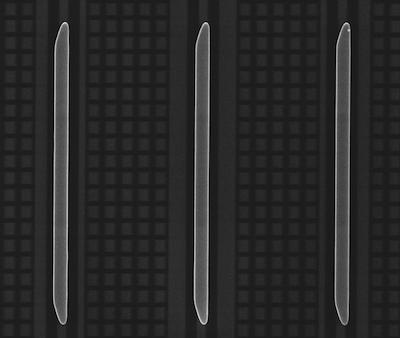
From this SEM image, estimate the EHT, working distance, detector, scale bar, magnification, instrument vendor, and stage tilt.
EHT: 18 kV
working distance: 5 mm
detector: TLD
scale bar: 20000 nm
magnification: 1.75 K X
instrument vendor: FEI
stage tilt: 0°